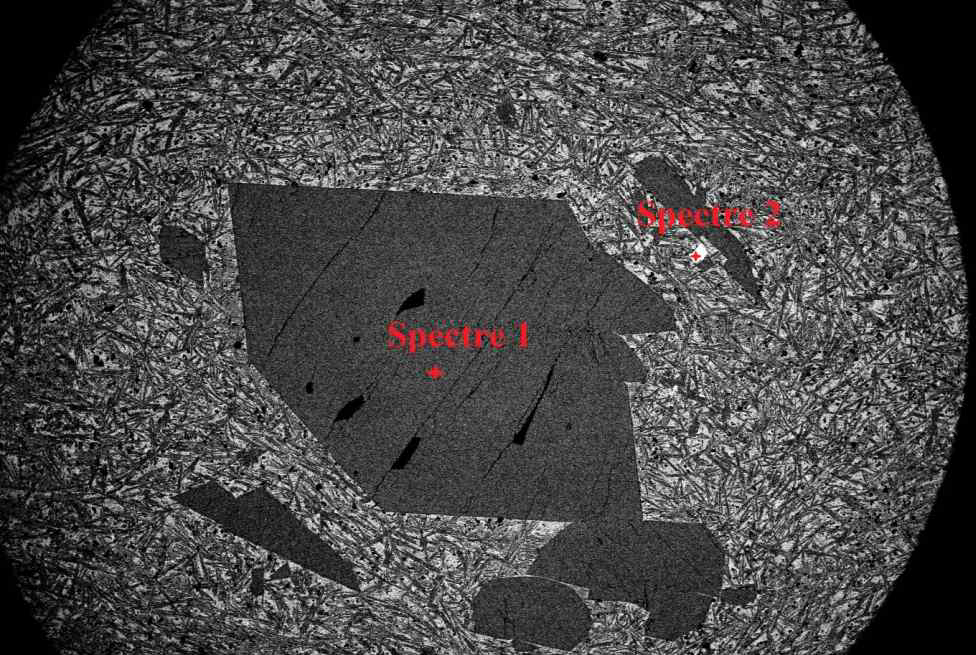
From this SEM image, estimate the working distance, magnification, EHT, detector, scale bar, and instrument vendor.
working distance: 10 mm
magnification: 0.03 K X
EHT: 15 kV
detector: BEC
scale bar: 500000 nm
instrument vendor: JEOL